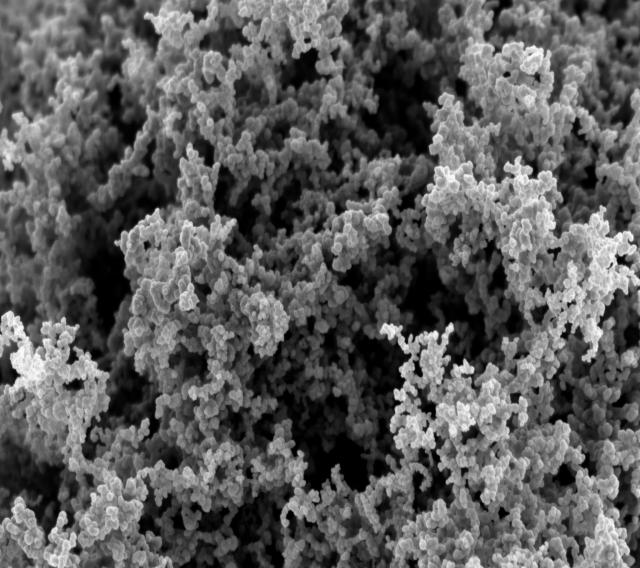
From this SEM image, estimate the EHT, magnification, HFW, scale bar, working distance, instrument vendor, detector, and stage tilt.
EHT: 2 kV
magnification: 20.03 K X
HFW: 5.709 µm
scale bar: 500 nm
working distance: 4 mm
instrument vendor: Zeiss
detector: InLens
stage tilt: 0°